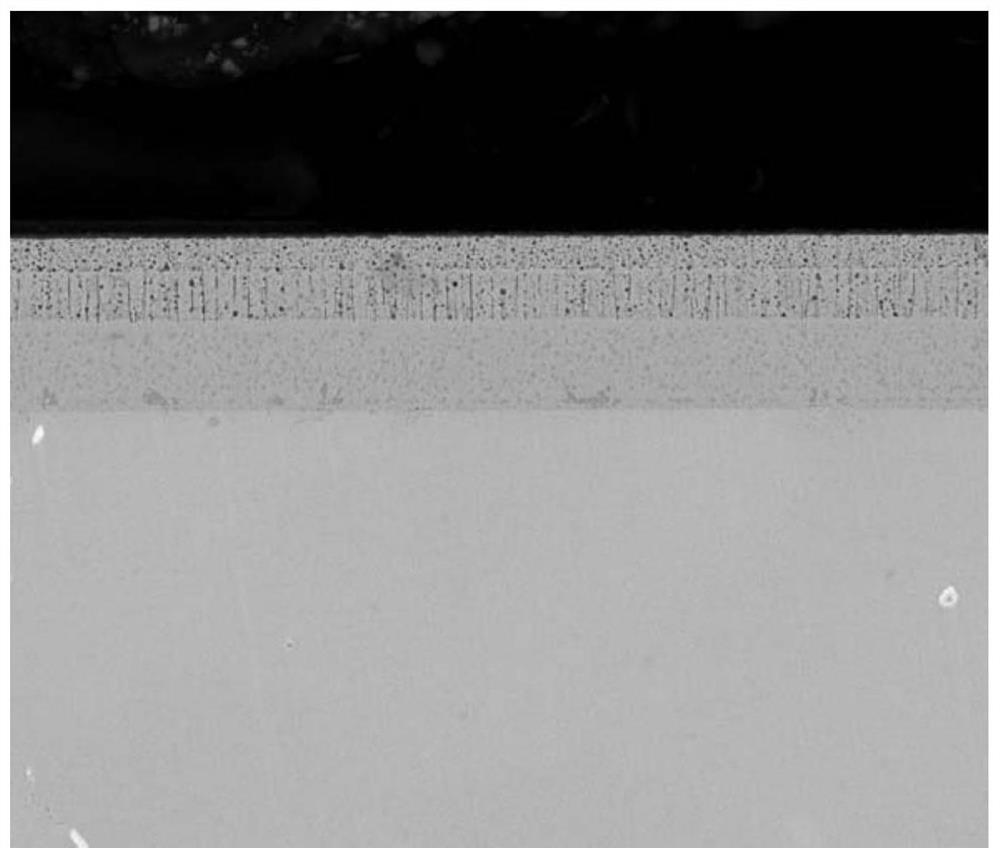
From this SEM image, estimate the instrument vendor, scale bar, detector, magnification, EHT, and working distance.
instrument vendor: FEI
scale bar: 50000 nm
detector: A+B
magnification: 1.5 K X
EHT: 25 kV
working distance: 10.1 mm